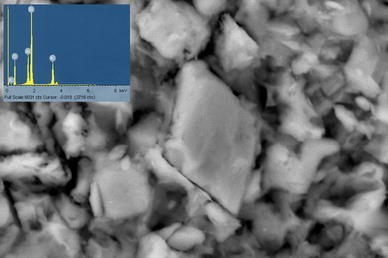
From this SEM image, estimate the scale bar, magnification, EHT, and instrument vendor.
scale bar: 5000 nm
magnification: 4.5 K X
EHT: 20 kV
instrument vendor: JEOL